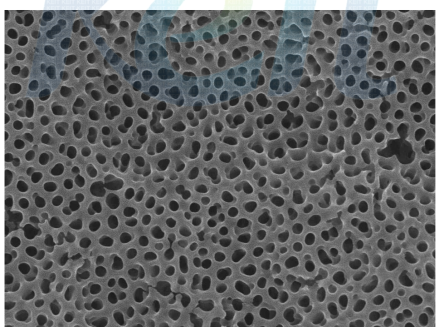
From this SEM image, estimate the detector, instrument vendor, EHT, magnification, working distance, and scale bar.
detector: SEI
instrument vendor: JEOL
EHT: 15 kV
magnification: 50 K X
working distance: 3 mm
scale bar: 100 nm